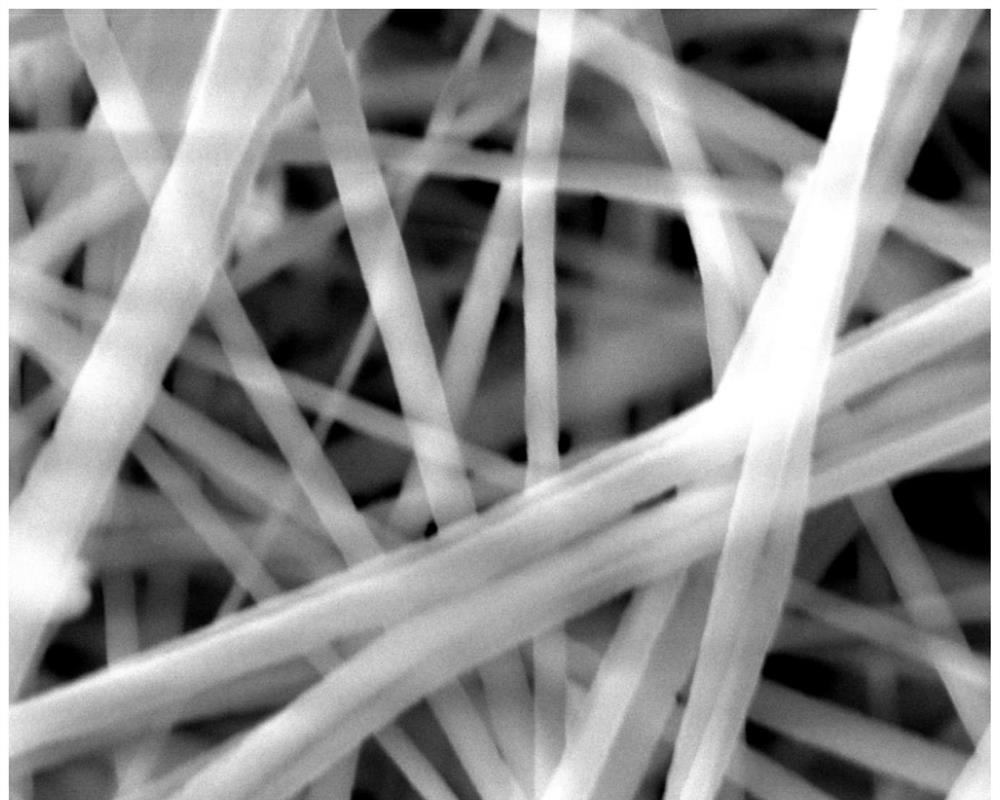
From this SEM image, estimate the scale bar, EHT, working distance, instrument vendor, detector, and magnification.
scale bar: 2000 nm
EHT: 20 kV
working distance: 11 mm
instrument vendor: FEI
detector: ETD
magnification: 50 K X